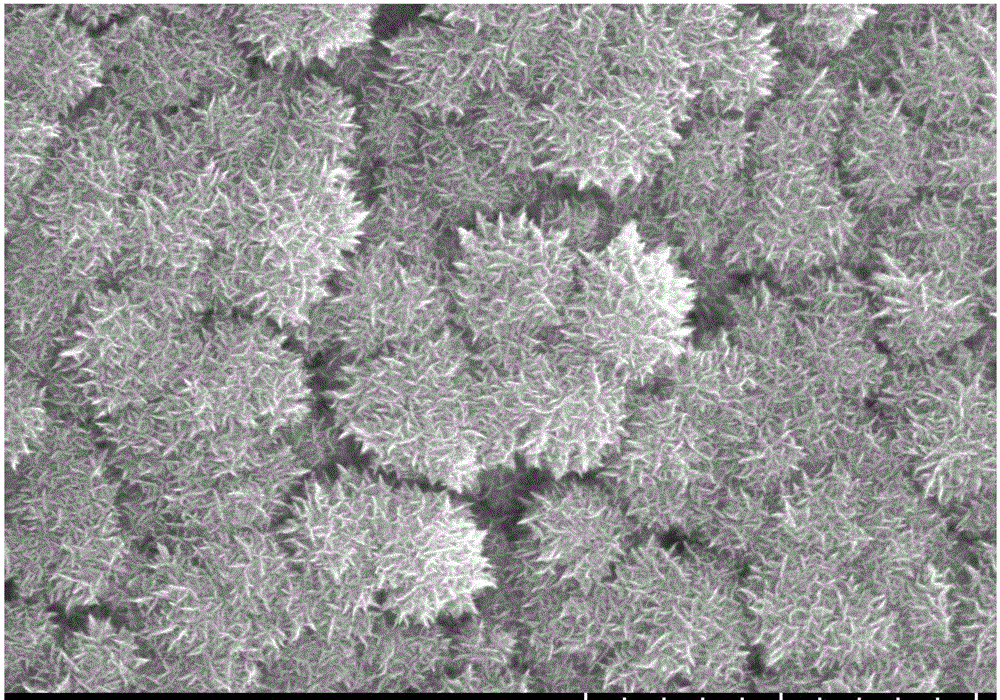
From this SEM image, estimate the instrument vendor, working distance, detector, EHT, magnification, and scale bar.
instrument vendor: Hitachi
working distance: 10.4 mm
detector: SE(U)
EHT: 10 kV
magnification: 50 K X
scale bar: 1000 nm